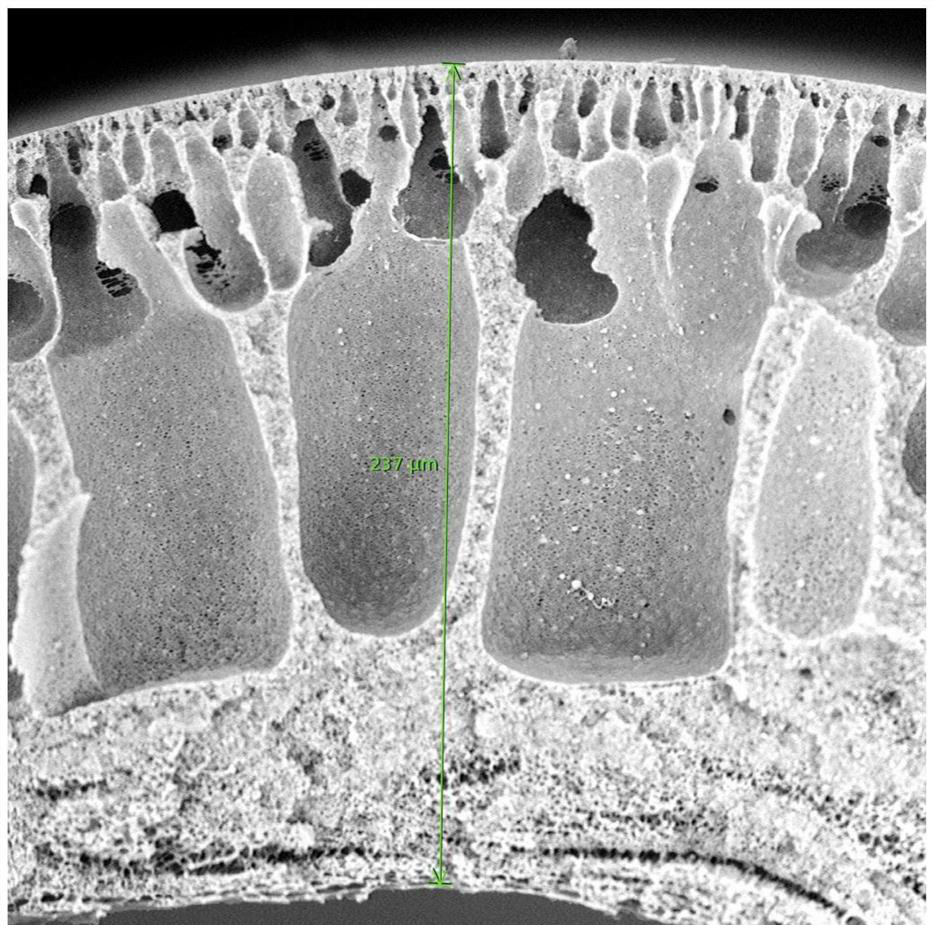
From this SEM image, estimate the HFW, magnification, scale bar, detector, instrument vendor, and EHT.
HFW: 268 µm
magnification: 1 K X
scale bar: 80000 nm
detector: BSD Full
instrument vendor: Thermo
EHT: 15 kV